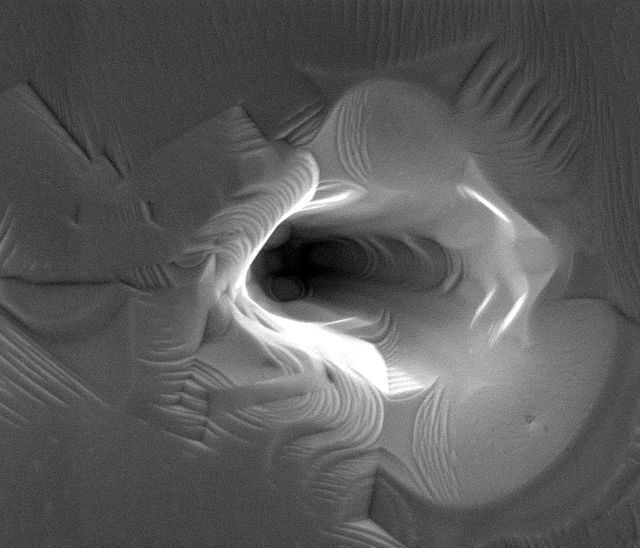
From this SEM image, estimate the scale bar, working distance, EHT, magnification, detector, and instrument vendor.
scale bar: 1000 nm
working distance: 5.4 mm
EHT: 5 kV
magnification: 24.999 K X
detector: ETD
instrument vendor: FEI